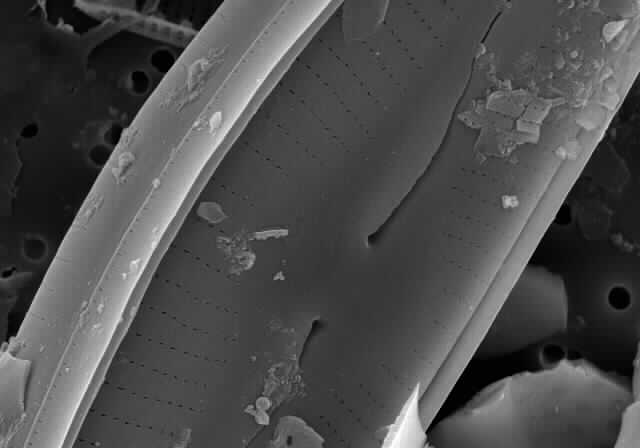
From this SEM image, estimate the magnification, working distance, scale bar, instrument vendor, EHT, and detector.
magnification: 8 K X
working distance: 14 mm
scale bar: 5000 nm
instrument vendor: Hitachi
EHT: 10 kV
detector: SE(M)